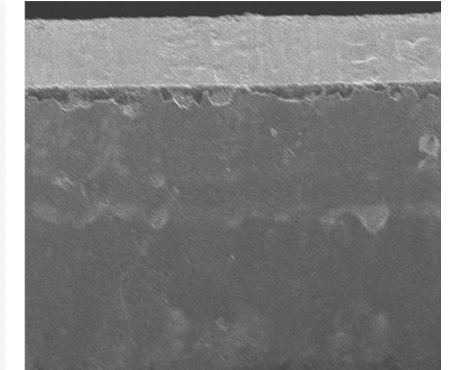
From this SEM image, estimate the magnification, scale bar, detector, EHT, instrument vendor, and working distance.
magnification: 1 K X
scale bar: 50000 nm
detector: SE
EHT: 10 kV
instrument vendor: Hitachi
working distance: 36.7 mm